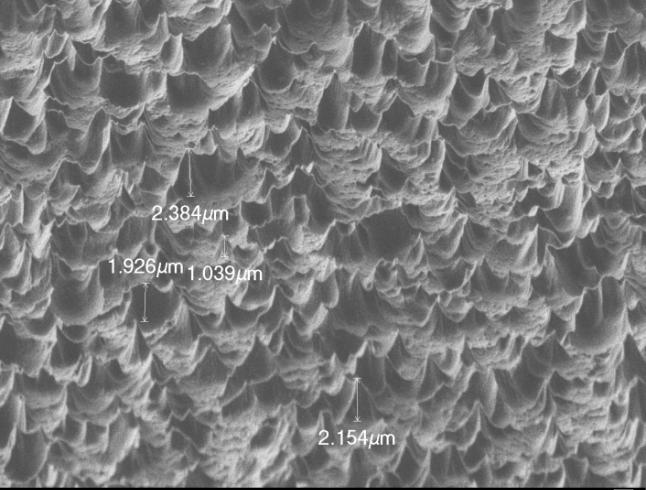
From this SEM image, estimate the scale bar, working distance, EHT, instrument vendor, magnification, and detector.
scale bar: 1000 nm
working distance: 6 mm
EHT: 1.5 kV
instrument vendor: JEOL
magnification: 3.7 K X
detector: LEI